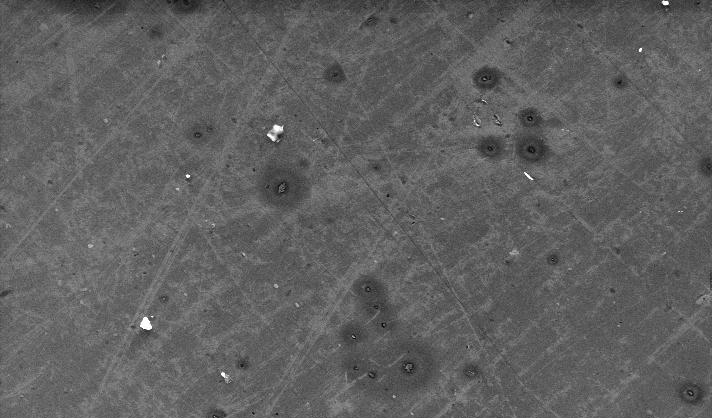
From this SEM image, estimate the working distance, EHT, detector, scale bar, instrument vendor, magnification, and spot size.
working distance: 10.1 mm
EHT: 20 kV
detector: SE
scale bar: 100000 nm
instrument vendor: FEI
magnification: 0.5 K X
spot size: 4.1